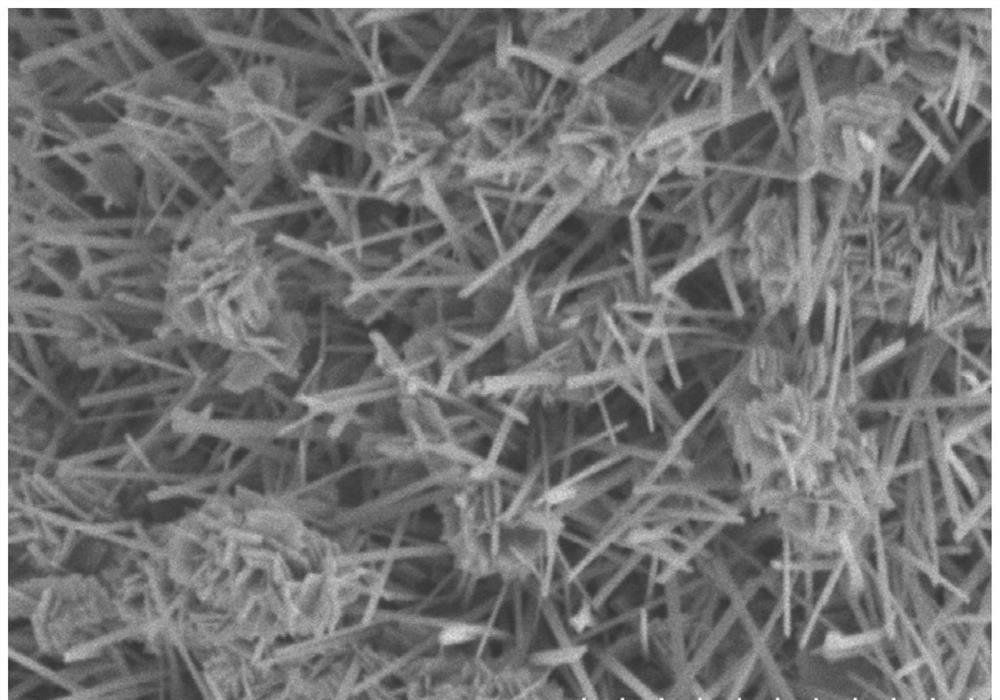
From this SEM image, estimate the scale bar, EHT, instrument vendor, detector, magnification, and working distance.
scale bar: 5000 nm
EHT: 5 kV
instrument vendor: Hitachi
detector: SE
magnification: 10 K X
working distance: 11.7 mm